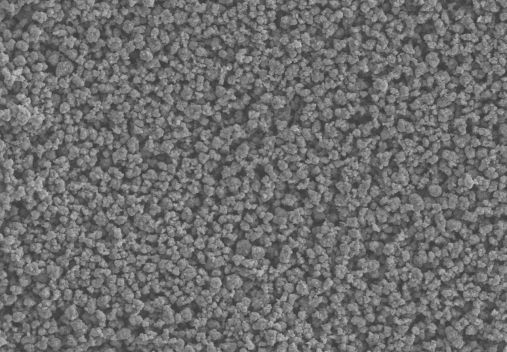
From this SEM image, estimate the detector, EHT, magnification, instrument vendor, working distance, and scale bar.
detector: SE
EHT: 15 kV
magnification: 1 K X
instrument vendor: Hitachi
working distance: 5.3 mm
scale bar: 50000 nm